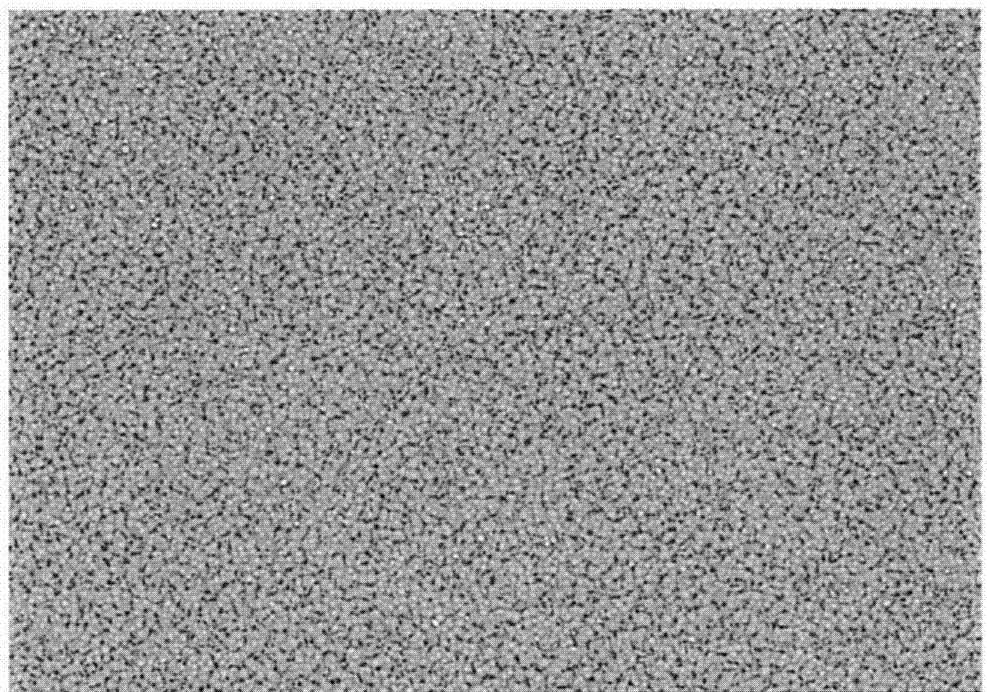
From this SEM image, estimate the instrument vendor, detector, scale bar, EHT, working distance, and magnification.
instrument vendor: JEOL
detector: BSE-COMP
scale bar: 200000 nm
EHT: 15 kV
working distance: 10.8 mm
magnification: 0.2 K X